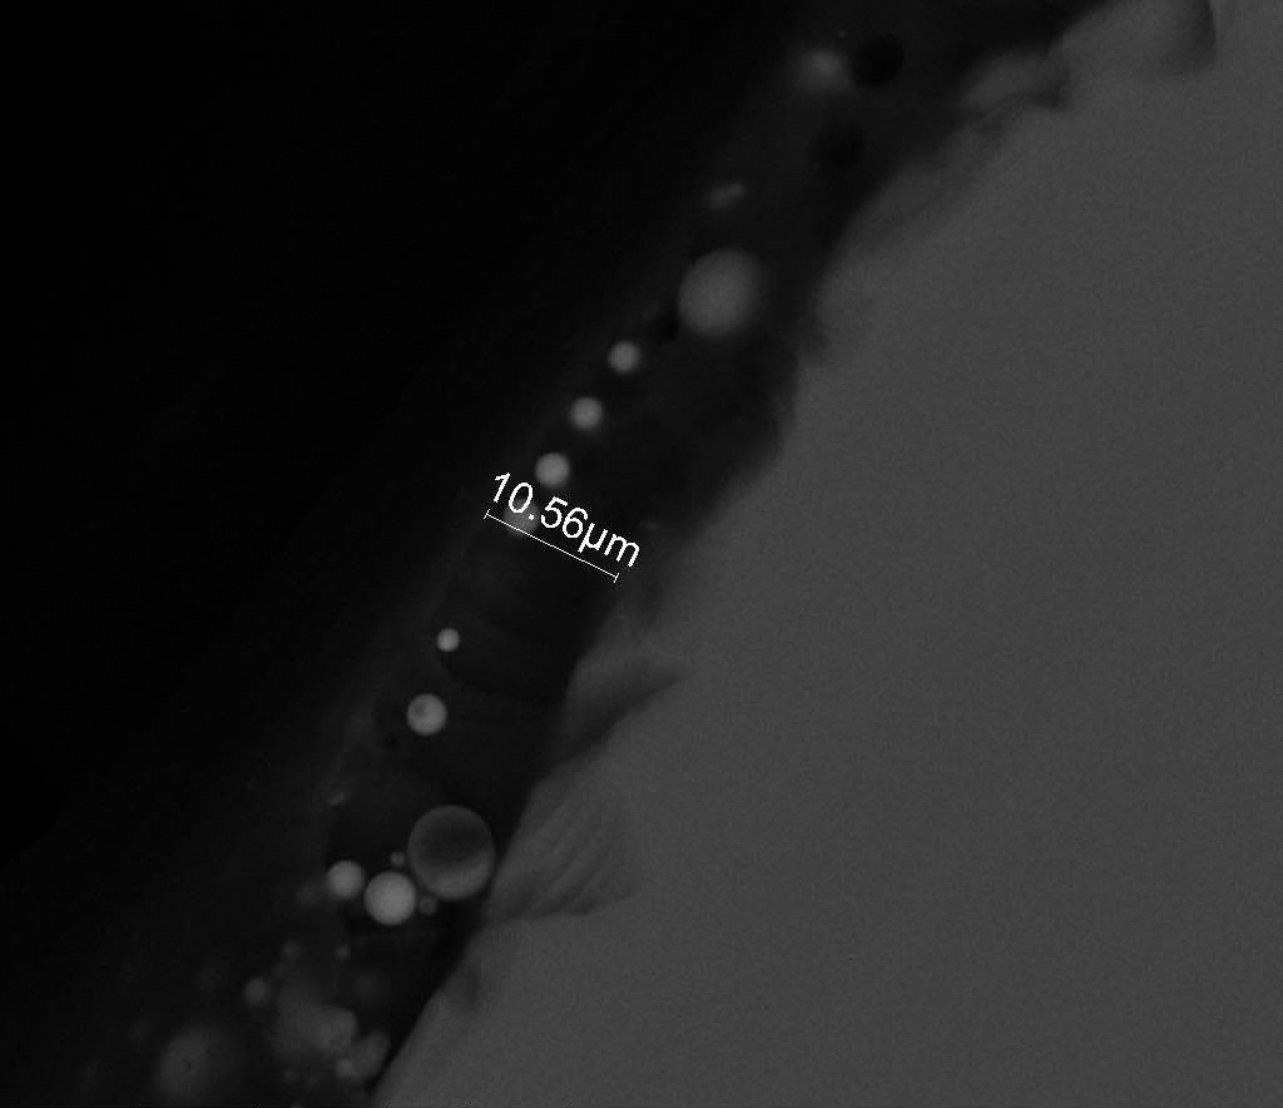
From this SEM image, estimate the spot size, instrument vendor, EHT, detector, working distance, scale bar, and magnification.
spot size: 4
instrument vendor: FEI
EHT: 25 kV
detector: A+B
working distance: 9.3 mm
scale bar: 50000 nm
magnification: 1.6 K X